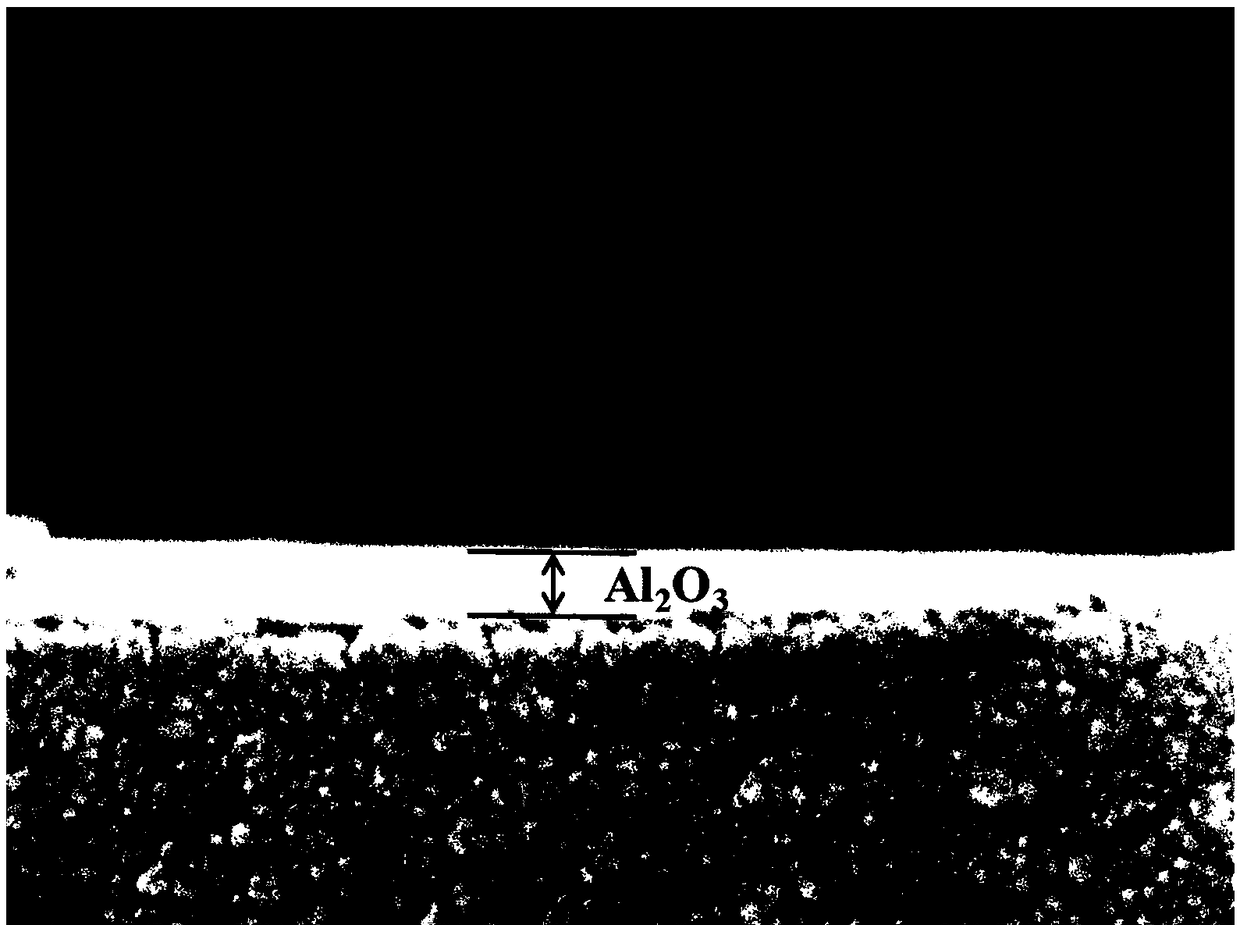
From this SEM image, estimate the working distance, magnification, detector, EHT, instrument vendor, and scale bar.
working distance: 9.6 mm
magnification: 100 K X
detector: SEI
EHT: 10 kV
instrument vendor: JEOL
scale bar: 100 nm